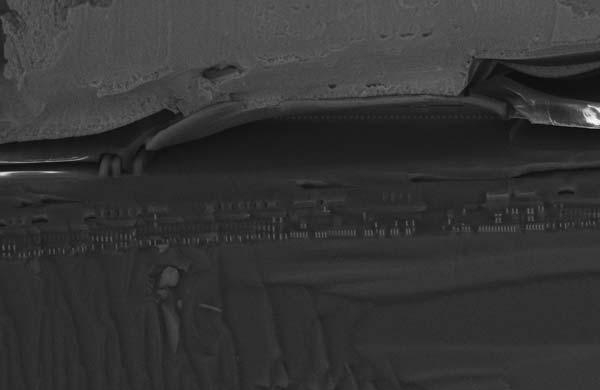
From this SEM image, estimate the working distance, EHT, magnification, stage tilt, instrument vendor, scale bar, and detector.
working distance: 4 mm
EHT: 15 kV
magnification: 3.16 K X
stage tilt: -15°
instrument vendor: Zeiss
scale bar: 10000 nm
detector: InLens